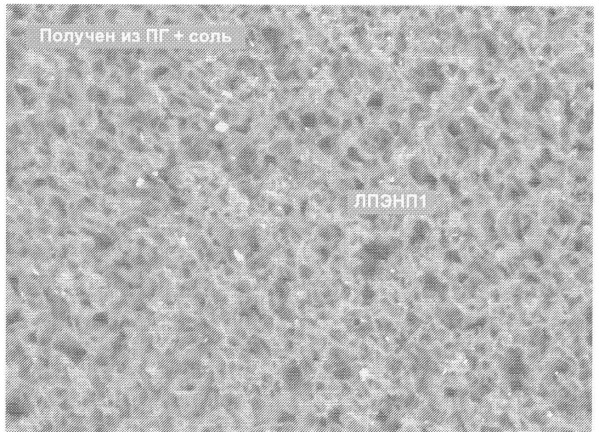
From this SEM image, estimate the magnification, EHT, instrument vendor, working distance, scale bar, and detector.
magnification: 1 K X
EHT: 10 kV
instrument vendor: JEOL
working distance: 15 mm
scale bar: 10000 nm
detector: COMPO